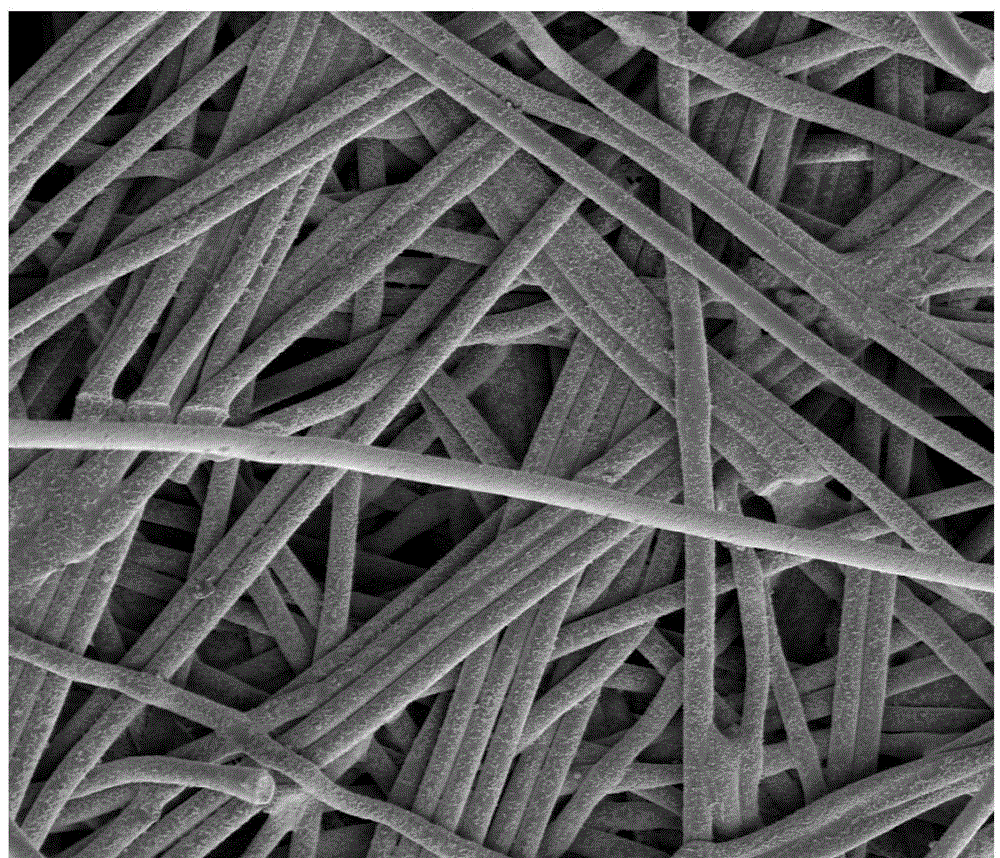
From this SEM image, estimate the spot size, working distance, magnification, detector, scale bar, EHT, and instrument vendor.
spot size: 3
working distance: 5.6 mm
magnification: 0.3 K X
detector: SE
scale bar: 100000 nm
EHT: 3 kV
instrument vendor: FEI